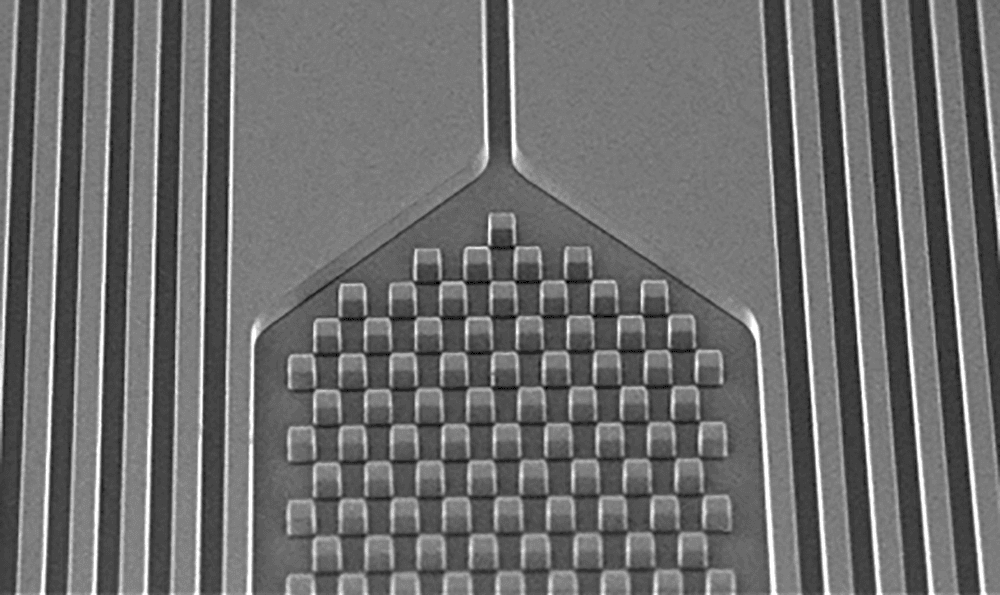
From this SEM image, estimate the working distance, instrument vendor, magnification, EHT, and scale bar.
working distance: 10.3 mm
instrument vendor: JEOL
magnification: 0.06 K X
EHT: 15 kV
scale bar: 500000 nm